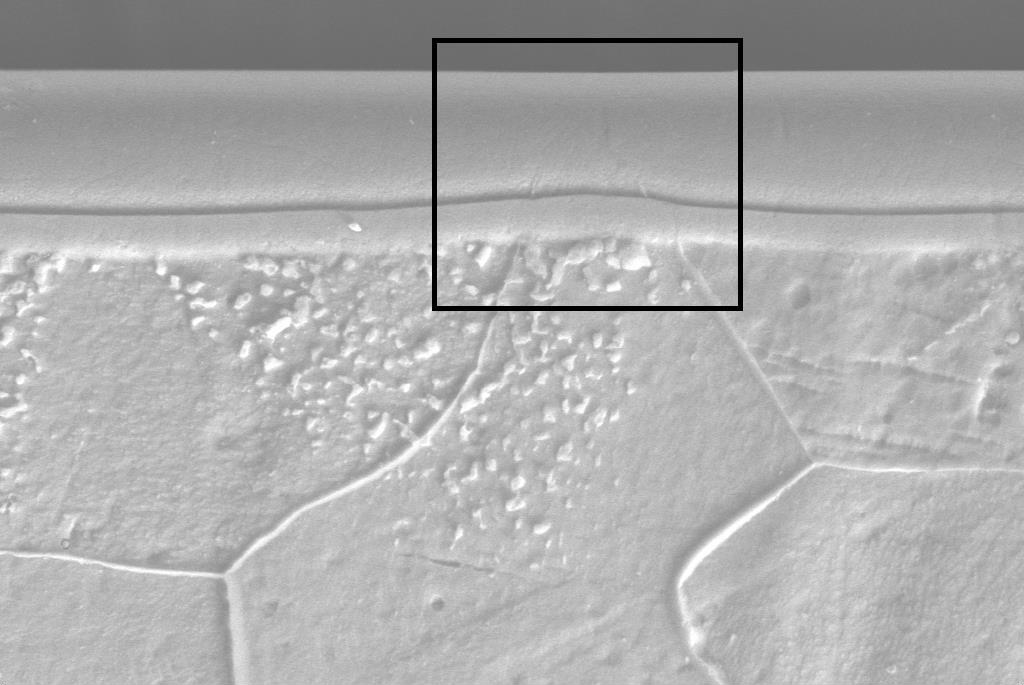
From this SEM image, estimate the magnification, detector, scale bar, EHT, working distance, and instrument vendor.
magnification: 5 K X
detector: SE1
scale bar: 2000 nm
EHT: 20 kV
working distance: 8.5 mm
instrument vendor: Zeiss